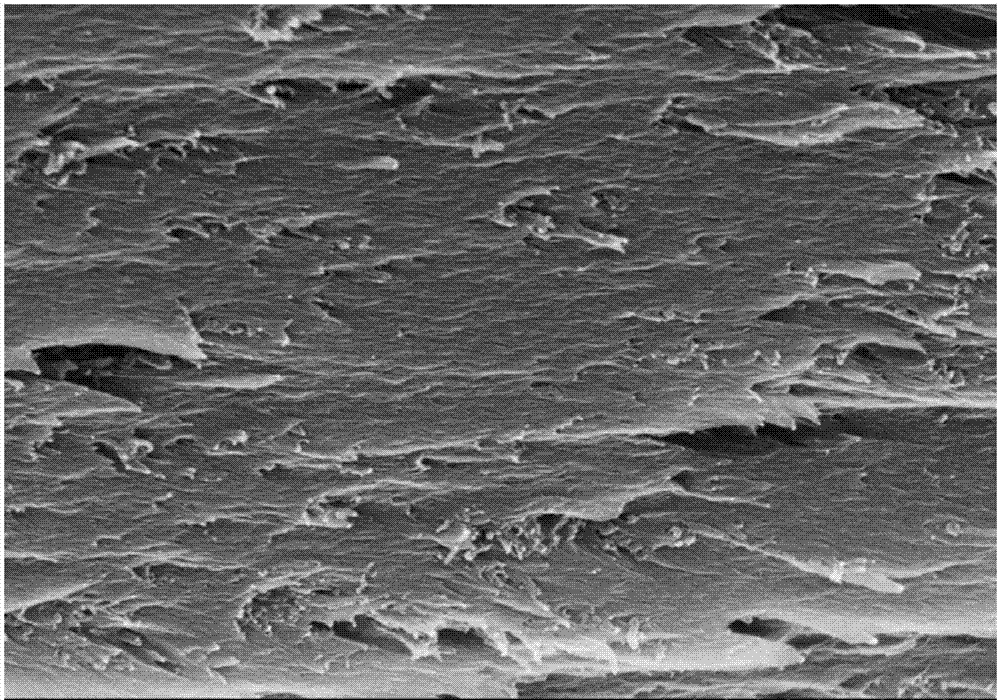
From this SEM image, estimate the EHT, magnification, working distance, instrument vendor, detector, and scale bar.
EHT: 15 kV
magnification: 20 K X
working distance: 16.7 mm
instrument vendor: Hitachi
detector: SE(U)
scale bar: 2000 nm